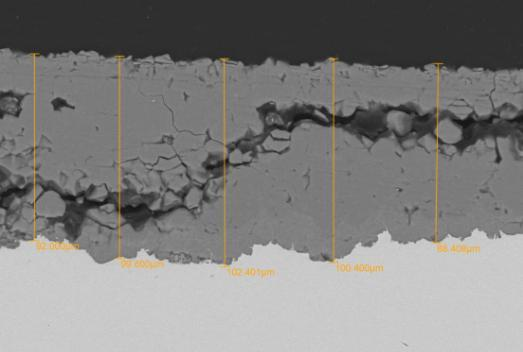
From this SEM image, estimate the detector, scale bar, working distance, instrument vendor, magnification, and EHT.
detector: BEC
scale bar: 50000 nm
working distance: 11 mm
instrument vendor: JEOL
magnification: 0.5 K X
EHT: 20 kV